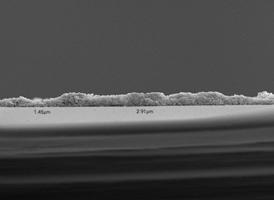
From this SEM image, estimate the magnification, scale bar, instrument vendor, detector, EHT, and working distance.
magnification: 2 K X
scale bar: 10000 nm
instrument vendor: JEOL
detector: SEI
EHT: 15 kV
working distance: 10.2 mm